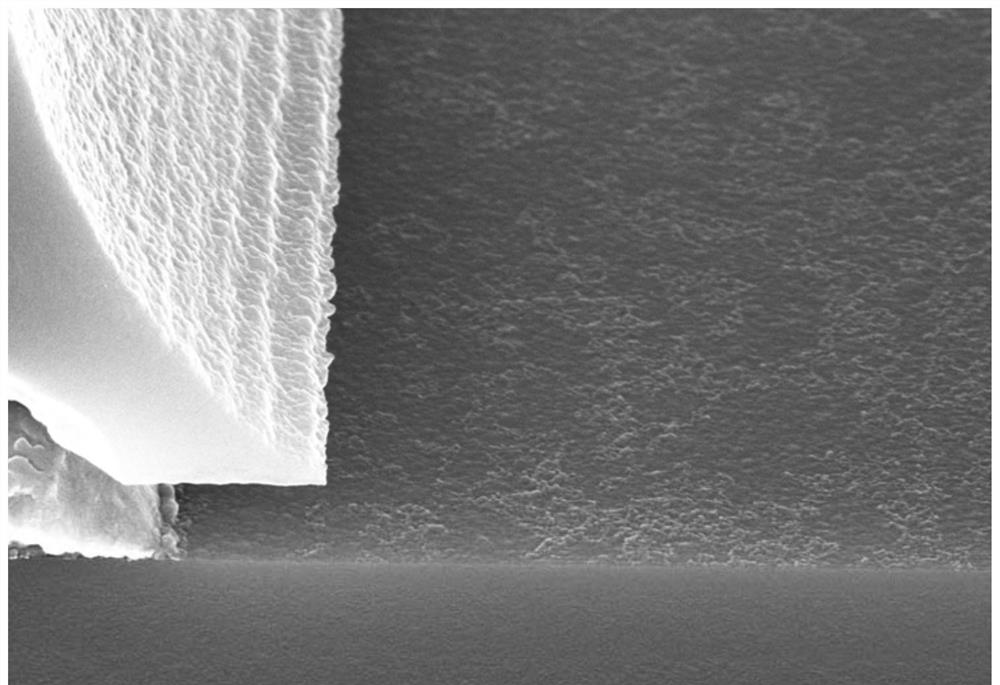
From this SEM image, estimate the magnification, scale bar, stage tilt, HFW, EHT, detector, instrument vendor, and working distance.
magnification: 30 K X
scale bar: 300 nm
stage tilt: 0°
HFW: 3.811 µm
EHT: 10 kV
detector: InLens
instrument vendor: Zeiss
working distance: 7.4 mm